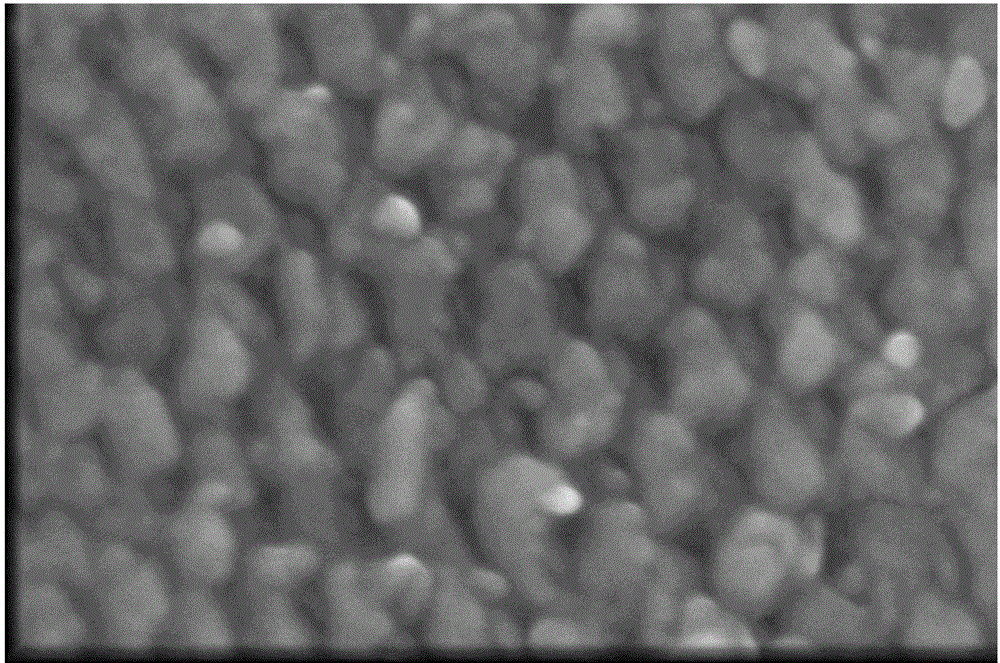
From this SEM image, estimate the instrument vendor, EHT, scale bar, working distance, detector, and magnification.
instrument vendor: FEI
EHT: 5 kV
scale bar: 300 nm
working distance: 6 mm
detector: TLD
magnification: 500 K X